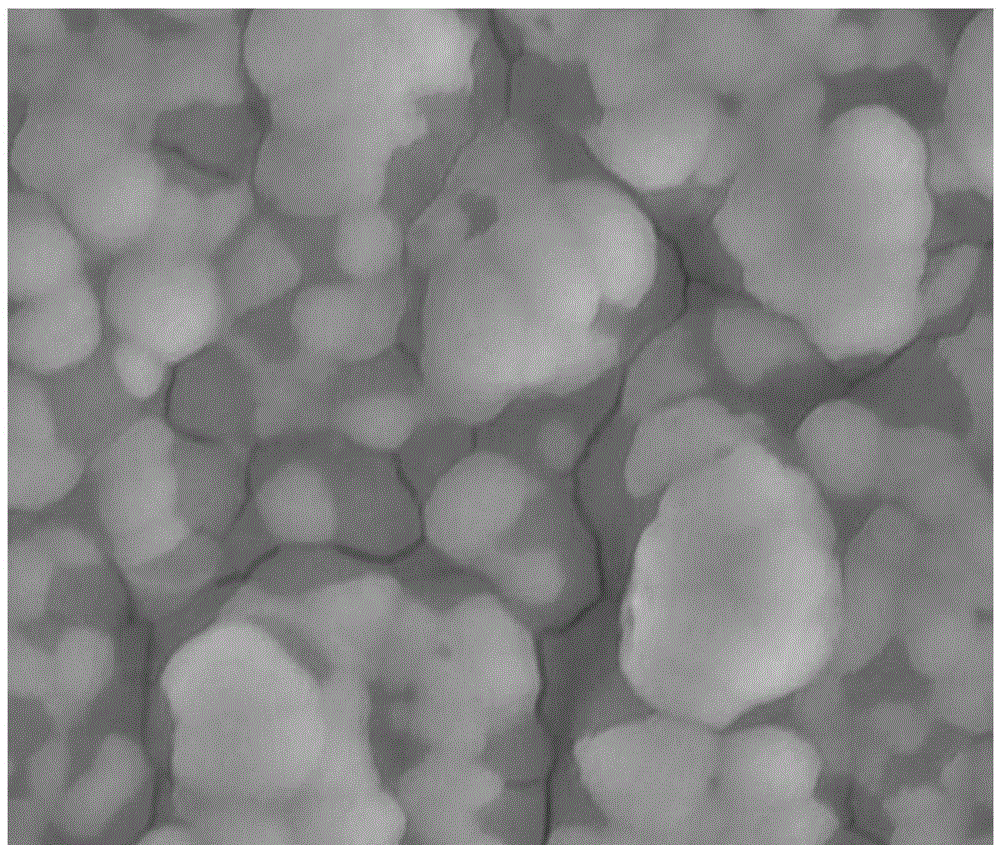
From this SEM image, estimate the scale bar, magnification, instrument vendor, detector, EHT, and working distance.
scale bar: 10000 nm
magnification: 10 K X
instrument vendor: FEI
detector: SE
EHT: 25 kV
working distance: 10.3 mm